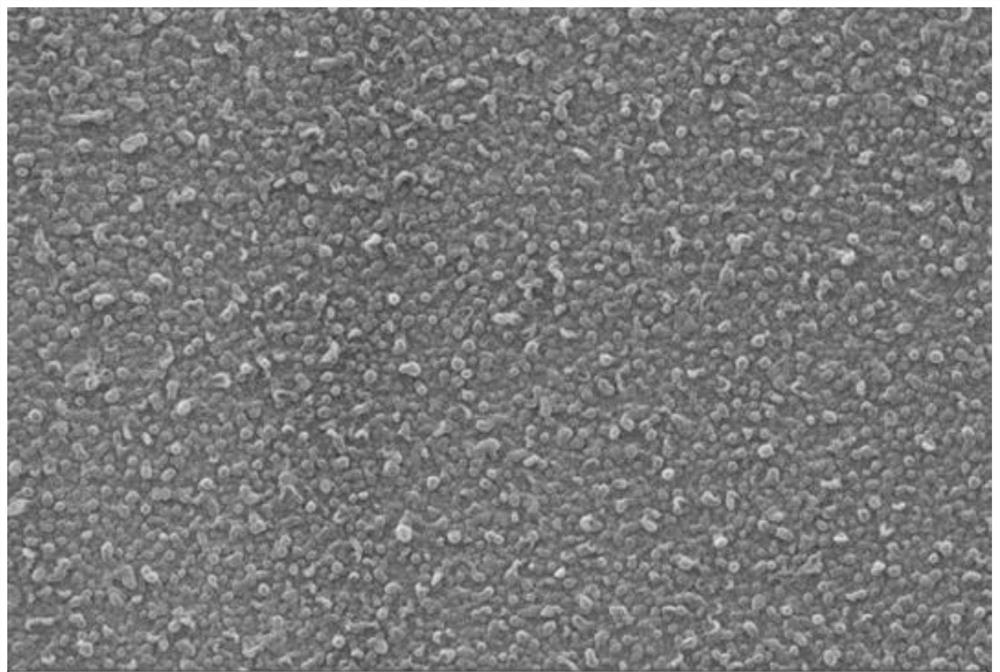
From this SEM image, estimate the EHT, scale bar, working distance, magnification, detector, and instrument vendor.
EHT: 20 kV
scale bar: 1000 nm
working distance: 8.5 mm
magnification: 10 K X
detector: SE1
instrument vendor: Zeiss